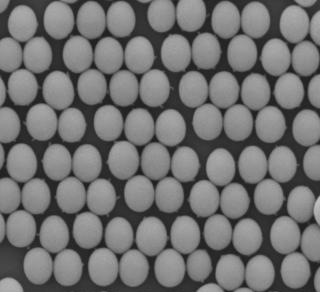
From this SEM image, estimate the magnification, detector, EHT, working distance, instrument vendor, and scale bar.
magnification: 30 K X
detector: LED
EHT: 5 kV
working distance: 8 mm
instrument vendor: JEOL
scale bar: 100 nm